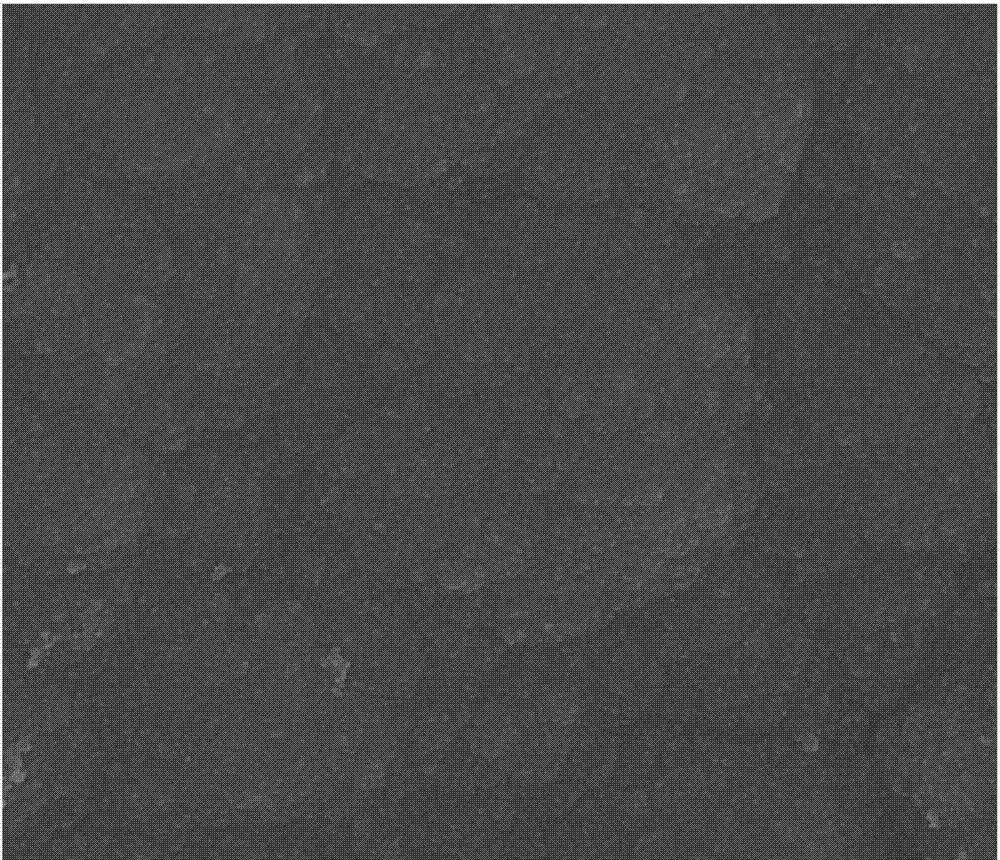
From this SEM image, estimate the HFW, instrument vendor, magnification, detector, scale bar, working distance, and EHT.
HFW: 4.8 µm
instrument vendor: FEI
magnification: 50 K X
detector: TLD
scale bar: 1000 nm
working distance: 5.3 mm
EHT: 10 kV